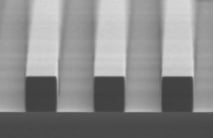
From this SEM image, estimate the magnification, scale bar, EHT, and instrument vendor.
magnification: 0.8 K X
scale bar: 50000 nm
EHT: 10 kV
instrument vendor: JEOL